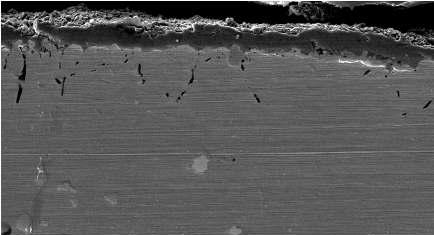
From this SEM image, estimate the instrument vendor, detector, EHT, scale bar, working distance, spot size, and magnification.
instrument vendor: FEI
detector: SE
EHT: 10 kV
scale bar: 20000 nm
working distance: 4.8 mm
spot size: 4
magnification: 4 K X